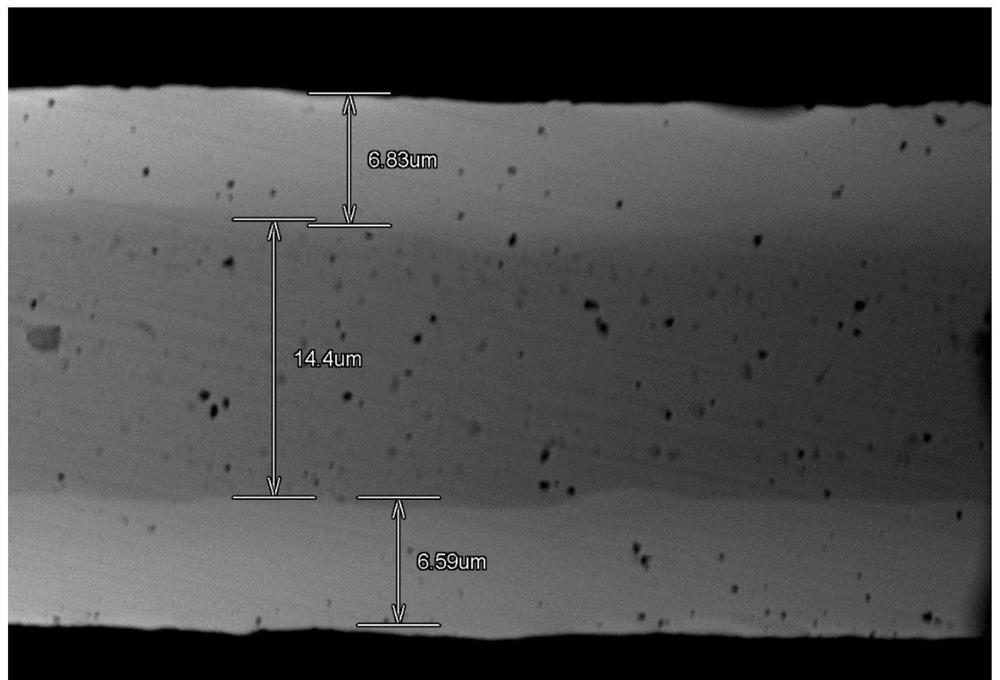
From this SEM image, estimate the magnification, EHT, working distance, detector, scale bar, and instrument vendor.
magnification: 2.5 K X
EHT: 15 kV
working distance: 14.4 mm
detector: BSECOMP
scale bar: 20000 nm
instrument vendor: Hitachi